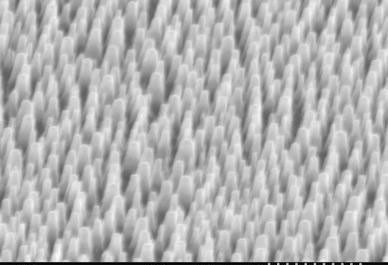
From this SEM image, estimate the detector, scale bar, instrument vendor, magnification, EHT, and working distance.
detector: SE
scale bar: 1000 nm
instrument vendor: Hitachi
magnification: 30 K X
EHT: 15 kV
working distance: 24 mm